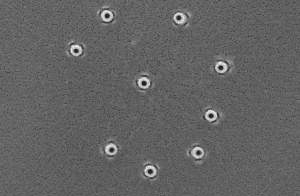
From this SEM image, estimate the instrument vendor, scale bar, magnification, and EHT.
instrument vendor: JEOL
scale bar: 1000 nm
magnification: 20 K X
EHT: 10 kV